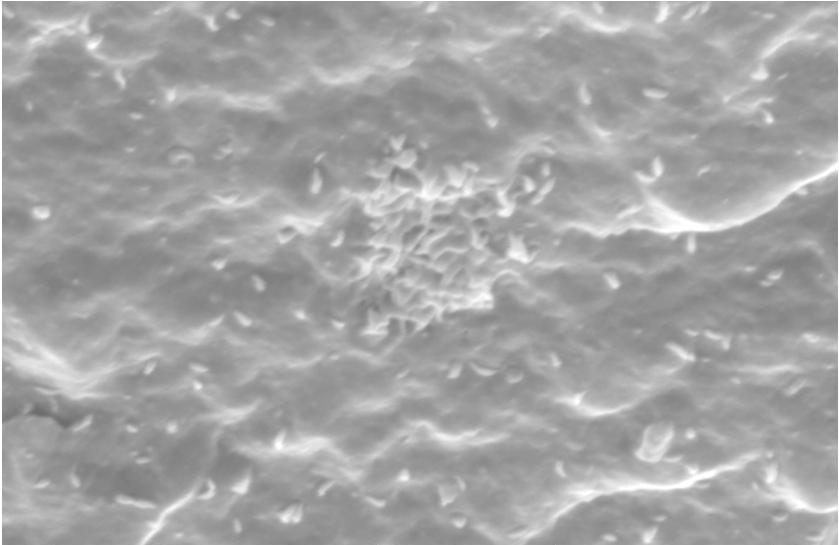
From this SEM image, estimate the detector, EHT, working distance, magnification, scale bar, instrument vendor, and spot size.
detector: SE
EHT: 15 kV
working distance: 21.1 mm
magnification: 2 K X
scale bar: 10000 nm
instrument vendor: FEI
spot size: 4.6